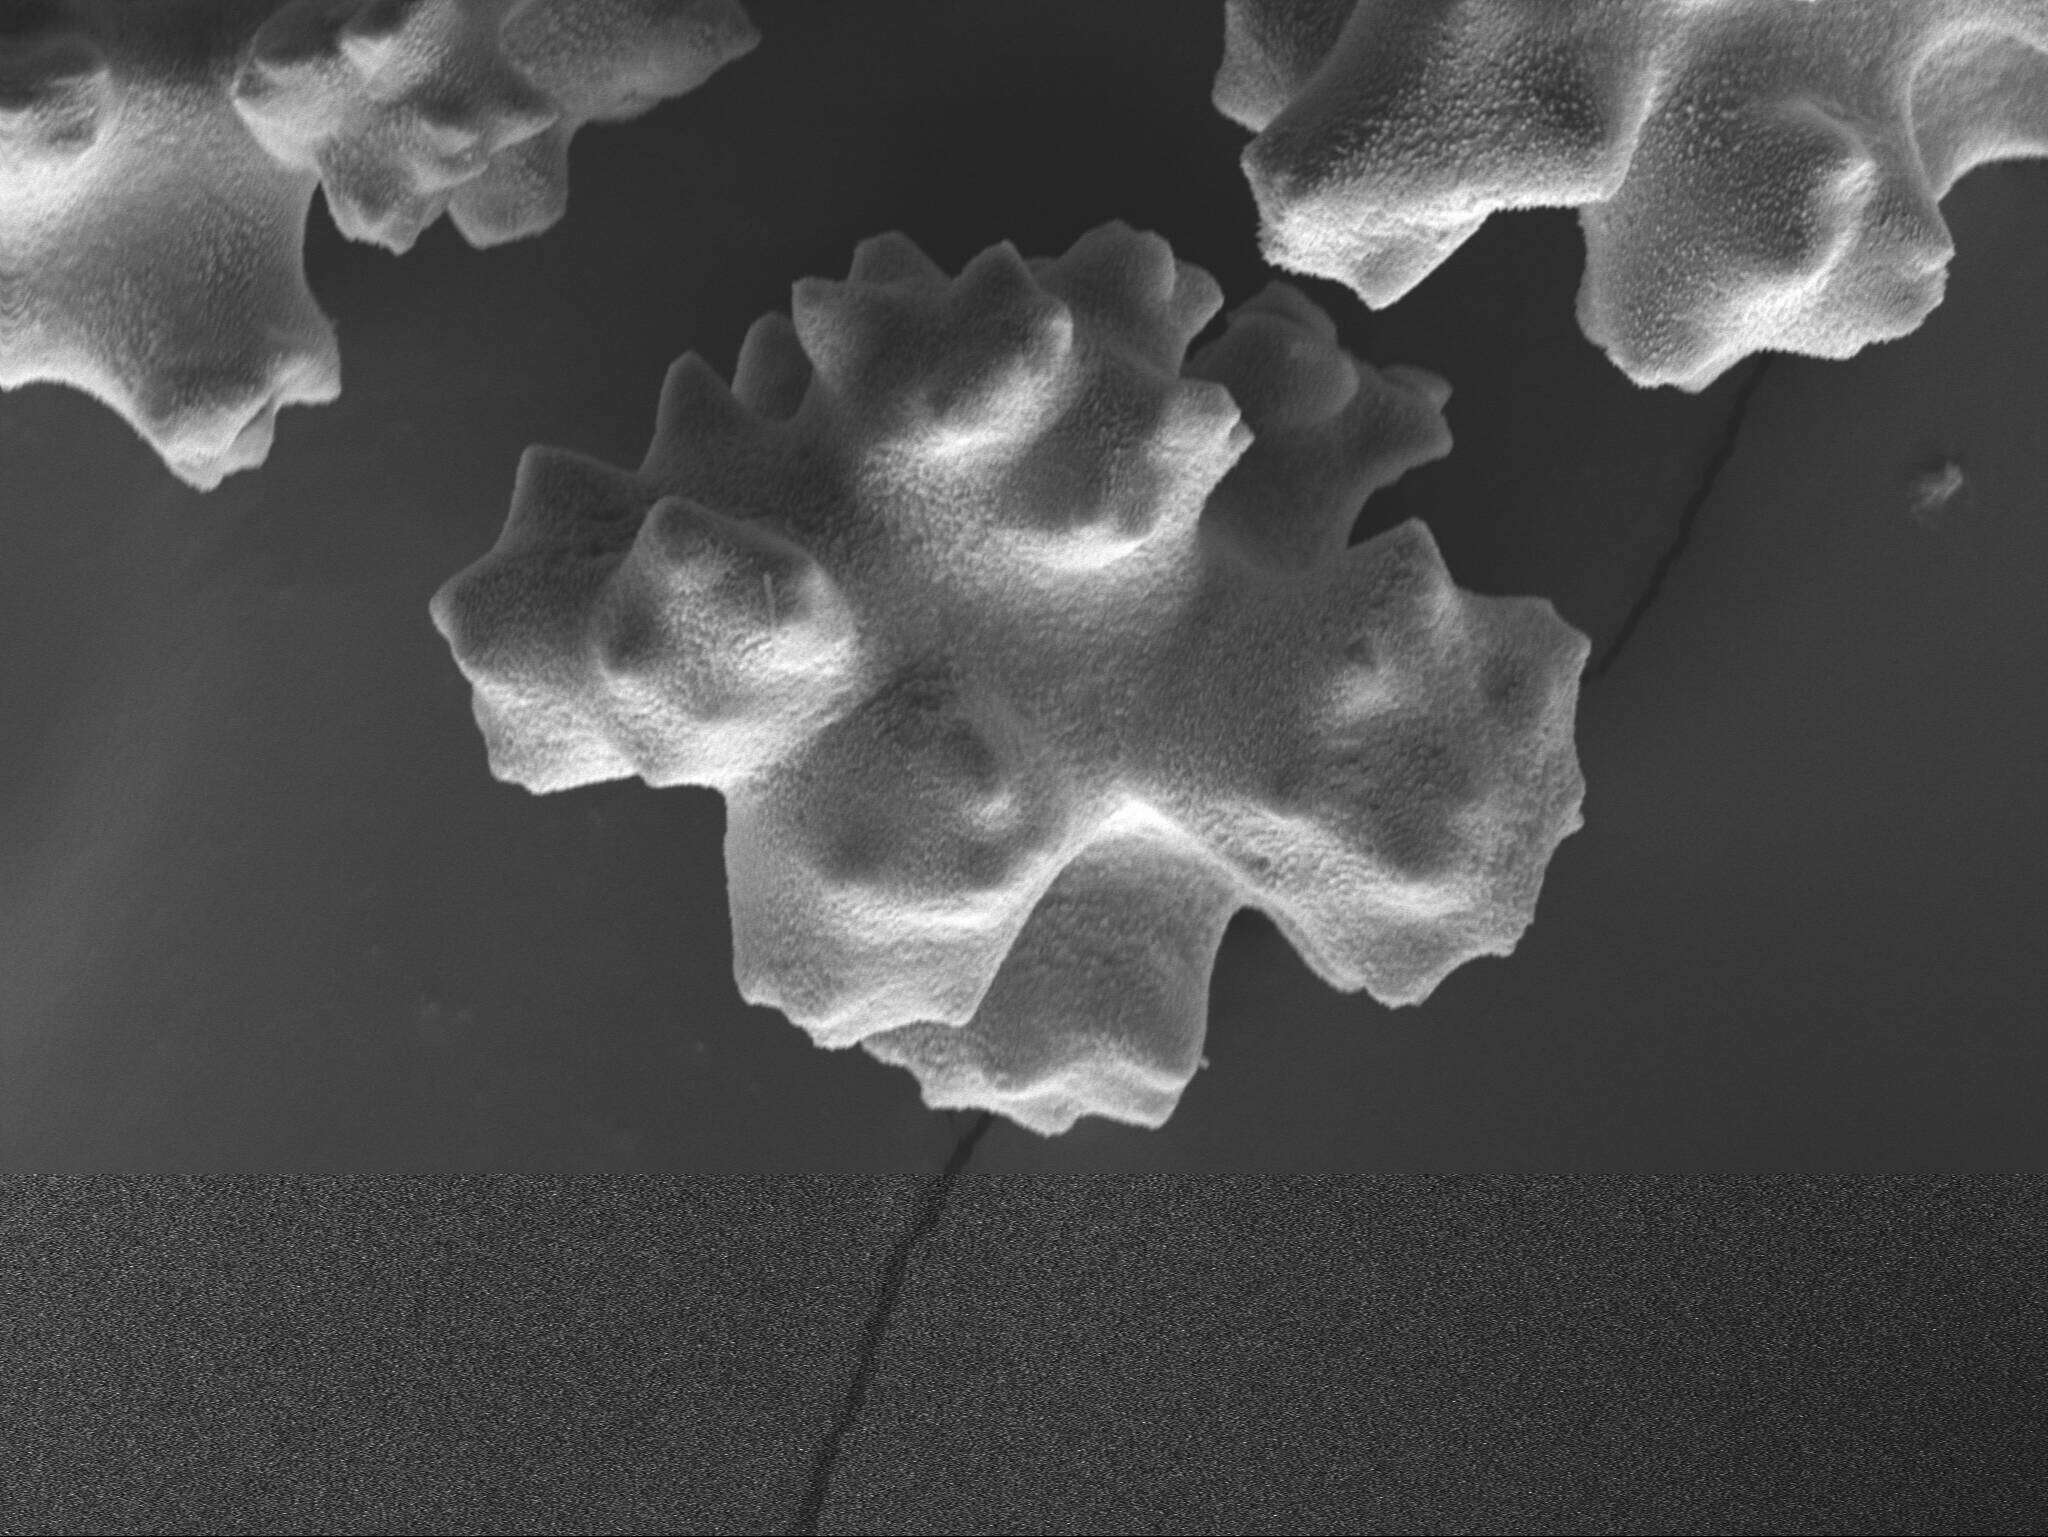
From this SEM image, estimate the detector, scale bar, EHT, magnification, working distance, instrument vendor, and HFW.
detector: SE1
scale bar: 20000 nm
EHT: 12 kV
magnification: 1 K X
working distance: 12.7 mm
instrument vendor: Zeiss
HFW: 114.4 µm